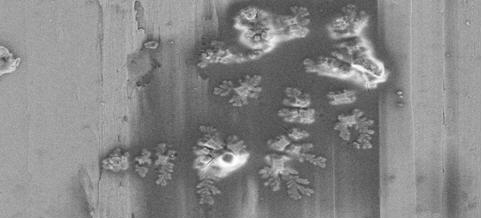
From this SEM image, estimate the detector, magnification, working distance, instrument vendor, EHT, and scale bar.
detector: SE2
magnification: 5 K X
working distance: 7.8 mm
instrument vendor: Zeiss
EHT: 5 kV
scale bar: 2000 nm